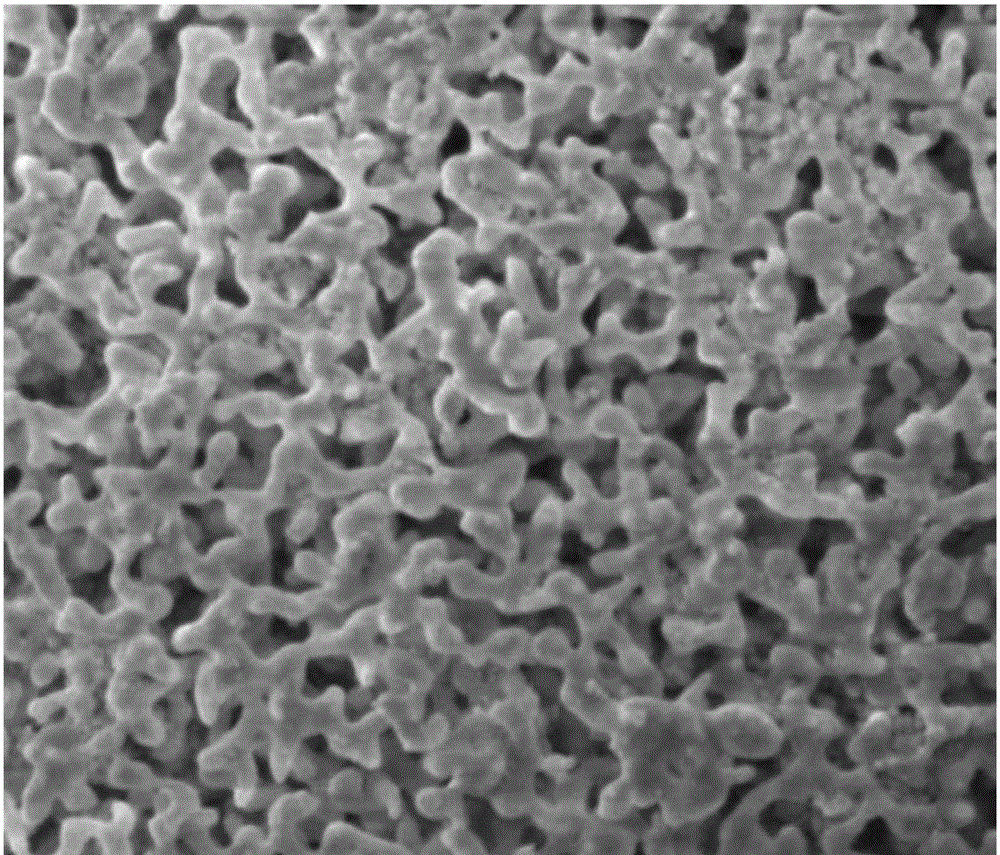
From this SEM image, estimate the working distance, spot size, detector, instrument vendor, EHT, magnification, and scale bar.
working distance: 6.3 mm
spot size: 4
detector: ETD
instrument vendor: FEI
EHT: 5 kV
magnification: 20 K X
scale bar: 5000 nm